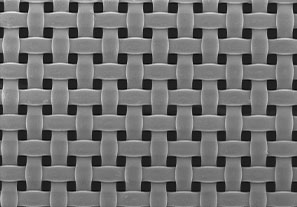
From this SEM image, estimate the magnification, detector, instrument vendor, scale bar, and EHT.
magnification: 0.1 K X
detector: SE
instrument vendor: Hitachi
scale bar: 500000 nm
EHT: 1 kV